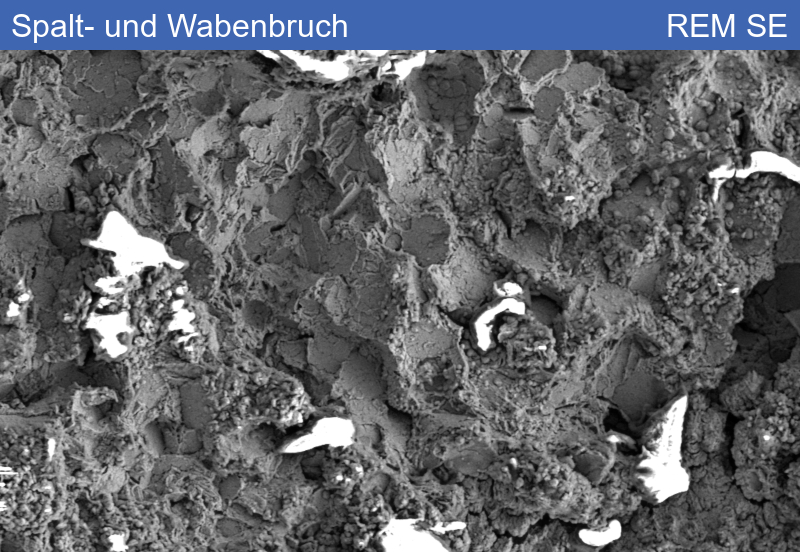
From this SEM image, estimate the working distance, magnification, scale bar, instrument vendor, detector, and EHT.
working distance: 8.42 mm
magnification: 0.5 K X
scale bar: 20000 nm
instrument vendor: Zeiss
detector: SE1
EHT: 10 kV